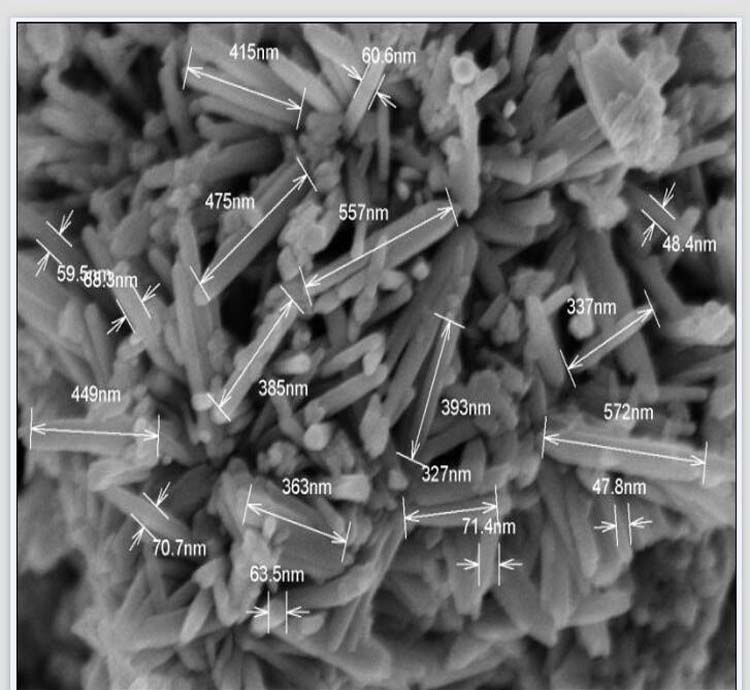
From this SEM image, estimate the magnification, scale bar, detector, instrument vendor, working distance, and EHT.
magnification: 50 K X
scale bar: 1000 nm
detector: SE(U)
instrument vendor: Hitachi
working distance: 8.8 mm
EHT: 5 kV